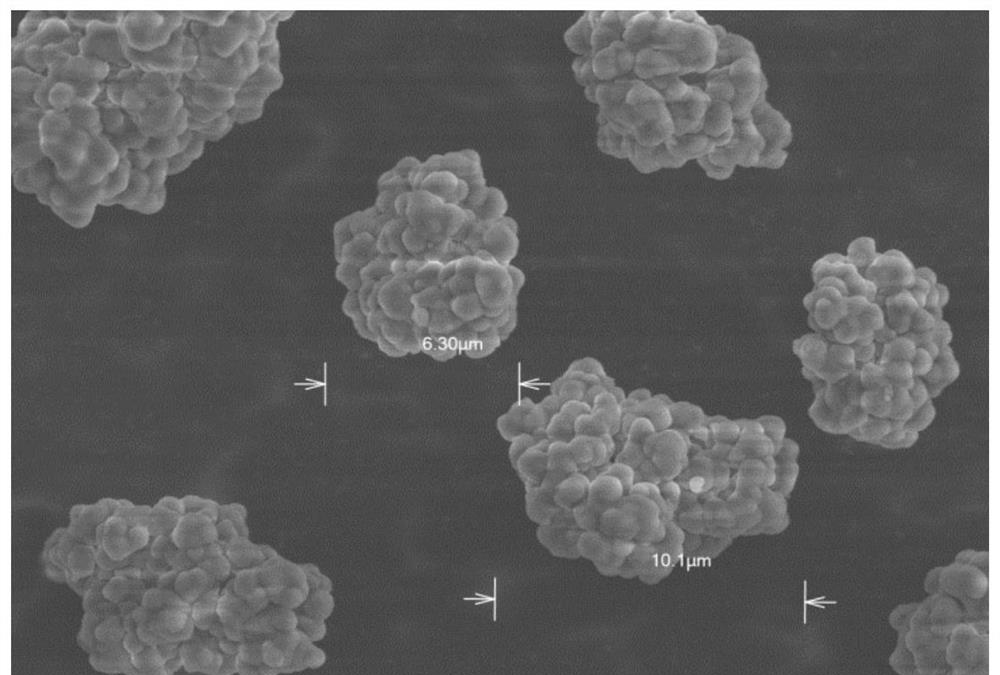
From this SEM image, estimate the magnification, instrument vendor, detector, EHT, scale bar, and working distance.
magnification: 4 K X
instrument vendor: Hitachi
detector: SE(UL)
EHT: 5 kV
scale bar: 10000 nm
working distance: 11.1 mm